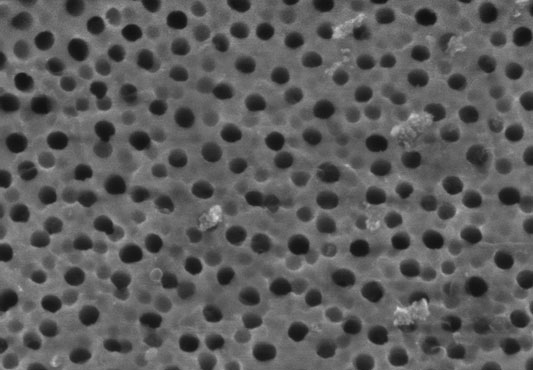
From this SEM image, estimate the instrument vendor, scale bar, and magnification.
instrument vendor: Hitachi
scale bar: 500 nm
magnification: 100 K X